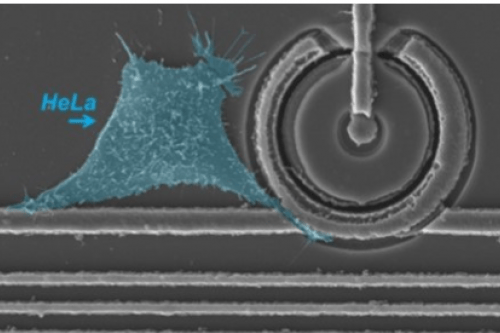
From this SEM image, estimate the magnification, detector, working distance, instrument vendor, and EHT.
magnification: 3 K X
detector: SE1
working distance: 7.5 mm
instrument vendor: Zeiss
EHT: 15 kV